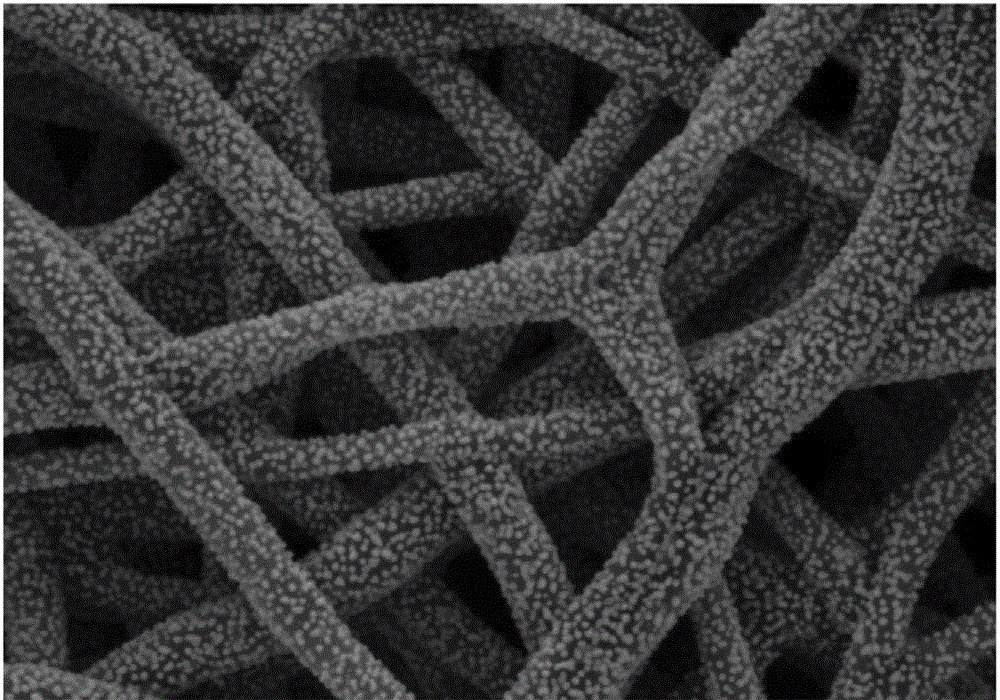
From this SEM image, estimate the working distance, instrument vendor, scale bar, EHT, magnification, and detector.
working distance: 9 mm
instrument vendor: Zeiss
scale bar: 1000 nm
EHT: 3 kV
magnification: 30 K X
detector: SE2